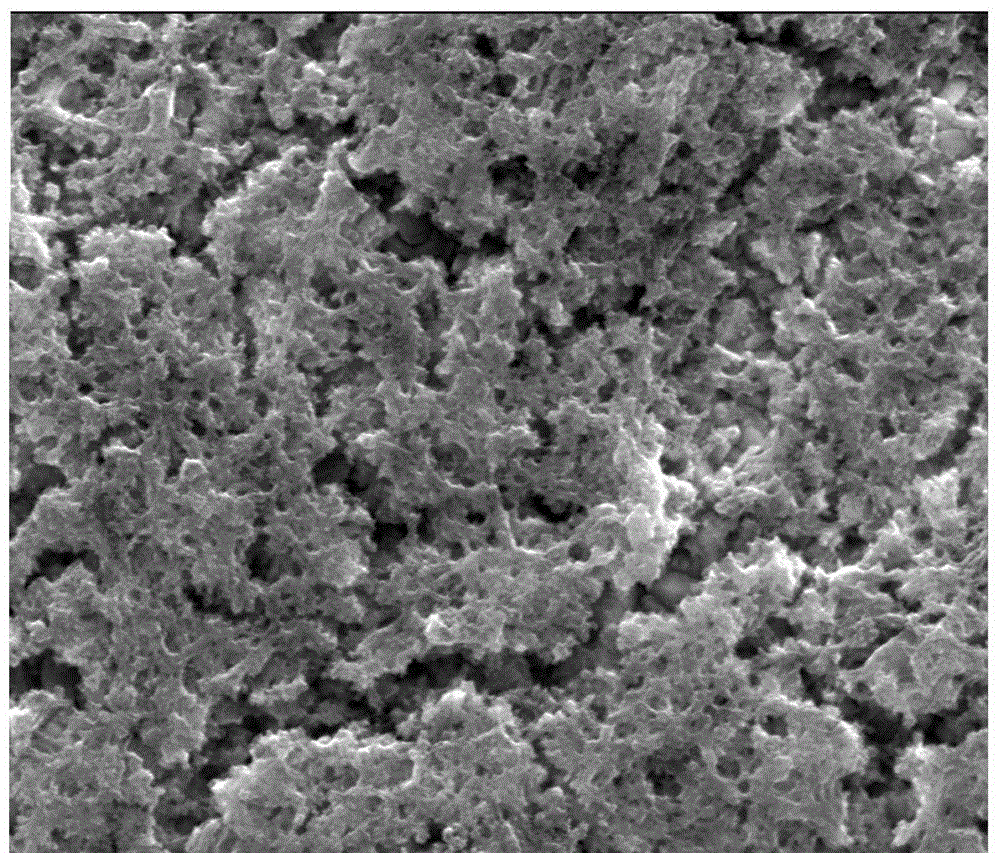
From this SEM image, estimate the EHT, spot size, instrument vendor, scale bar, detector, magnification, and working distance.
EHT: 20 kV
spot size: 3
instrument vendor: FEI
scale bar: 2000 nm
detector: ETD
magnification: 50 K X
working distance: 9.4 mm